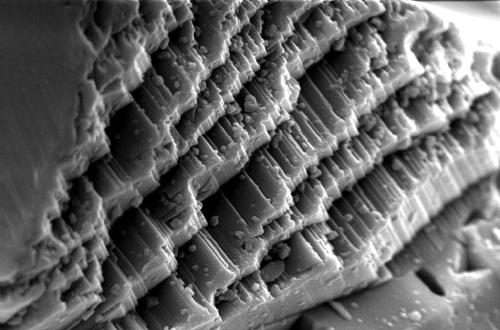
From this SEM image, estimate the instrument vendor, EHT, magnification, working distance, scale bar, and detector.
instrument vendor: Zeiss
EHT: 20 kV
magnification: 3 K X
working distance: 8.5 mm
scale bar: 2000 nm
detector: SE1